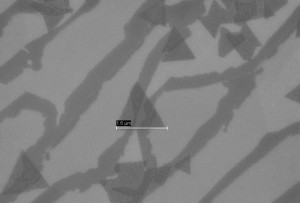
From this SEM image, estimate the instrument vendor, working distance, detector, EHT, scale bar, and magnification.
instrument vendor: Zeiss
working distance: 11 mm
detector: InLens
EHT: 5 kV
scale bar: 2000 nm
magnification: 11.03 K X